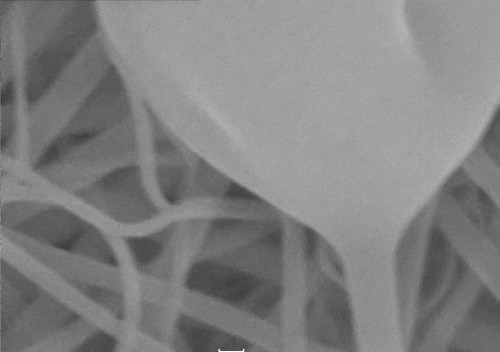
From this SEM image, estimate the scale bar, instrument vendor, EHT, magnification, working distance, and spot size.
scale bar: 100 nm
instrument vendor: other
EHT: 20 kV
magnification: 100 K X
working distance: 5.9 mm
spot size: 5